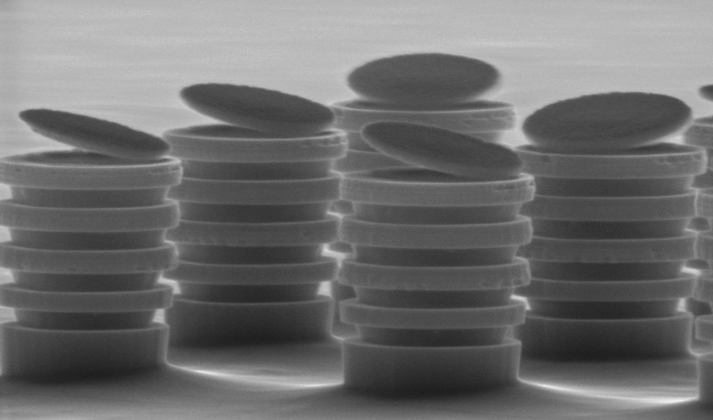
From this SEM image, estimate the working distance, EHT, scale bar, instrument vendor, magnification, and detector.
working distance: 9.8 mm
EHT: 20 kV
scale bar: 2000 nm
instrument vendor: FEI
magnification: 13.633 K X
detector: SE